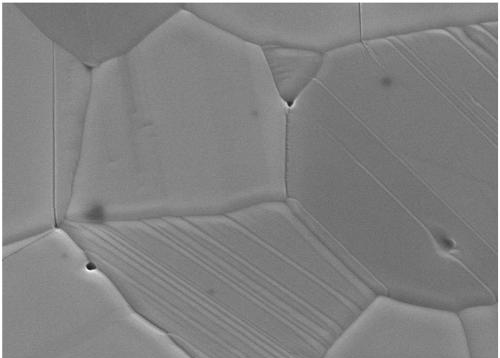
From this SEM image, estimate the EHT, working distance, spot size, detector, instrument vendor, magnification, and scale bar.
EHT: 20 kV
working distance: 12.3 mm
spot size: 3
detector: ETD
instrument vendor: FEI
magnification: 30 K X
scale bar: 4000 nm